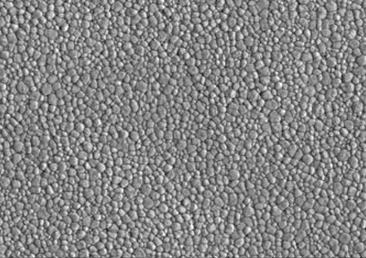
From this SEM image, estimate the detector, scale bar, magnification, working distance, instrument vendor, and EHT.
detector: SE(L)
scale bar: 10000 nm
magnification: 5 K X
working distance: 15.3 mm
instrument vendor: Hitachi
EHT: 10 kV